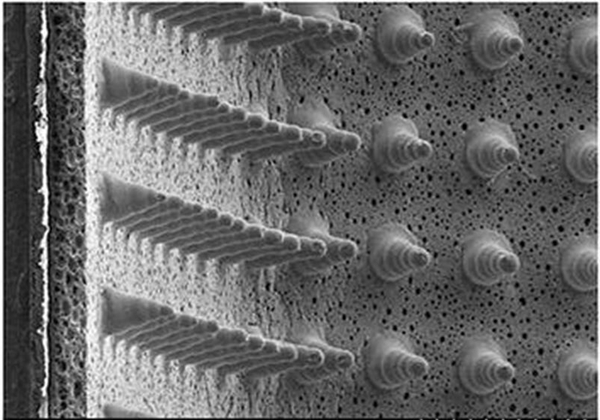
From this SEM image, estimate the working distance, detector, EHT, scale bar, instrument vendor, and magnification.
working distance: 7.7 mm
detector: SE(M)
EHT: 1 kV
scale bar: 1000000 nm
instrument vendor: Hitachi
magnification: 0.035 K X